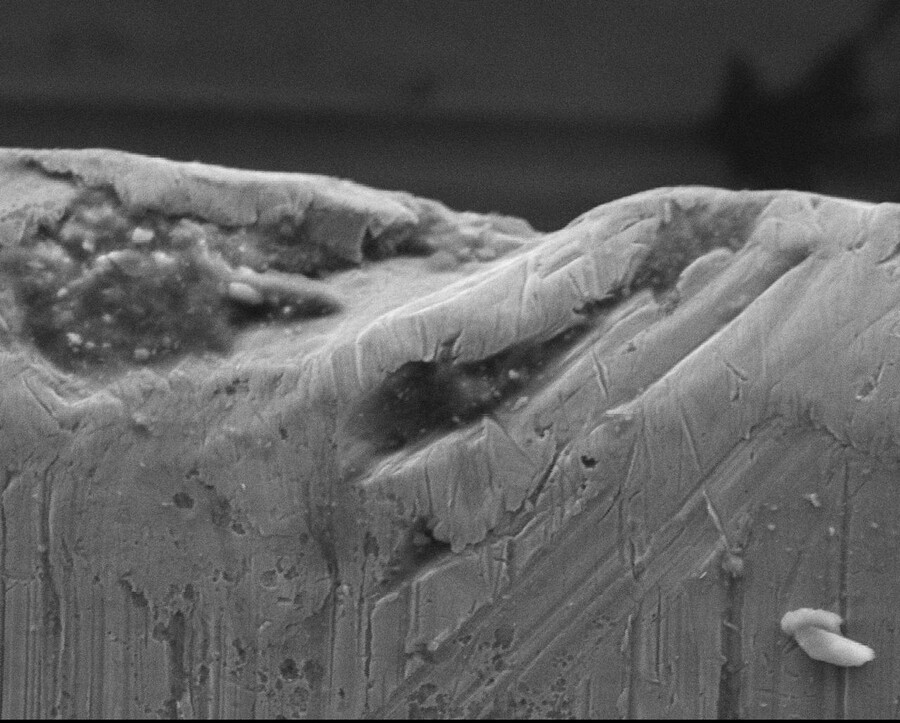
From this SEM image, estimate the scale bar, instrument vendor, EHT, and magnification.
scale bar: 20000 nm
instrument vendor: JEOL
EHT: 15 kV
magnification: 0.9 K X